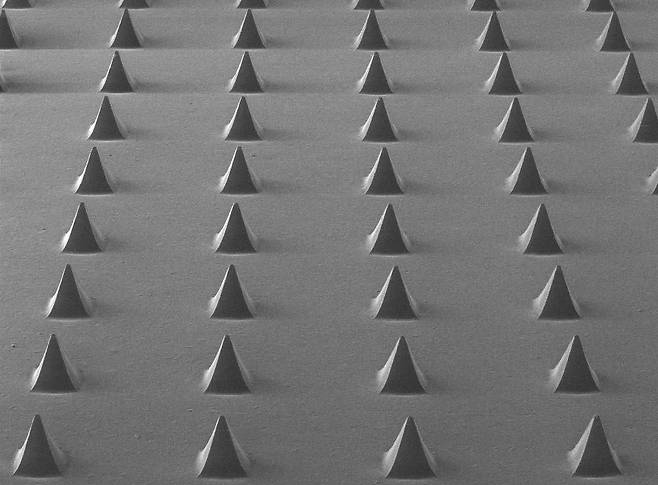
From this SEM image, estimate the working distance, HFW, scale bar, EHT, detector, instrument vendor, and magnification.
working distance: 9.08 mm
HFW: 2540 µm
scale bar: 500000 nm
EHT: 10 kV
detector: SE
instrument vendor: Tescan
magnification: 0.1 K X